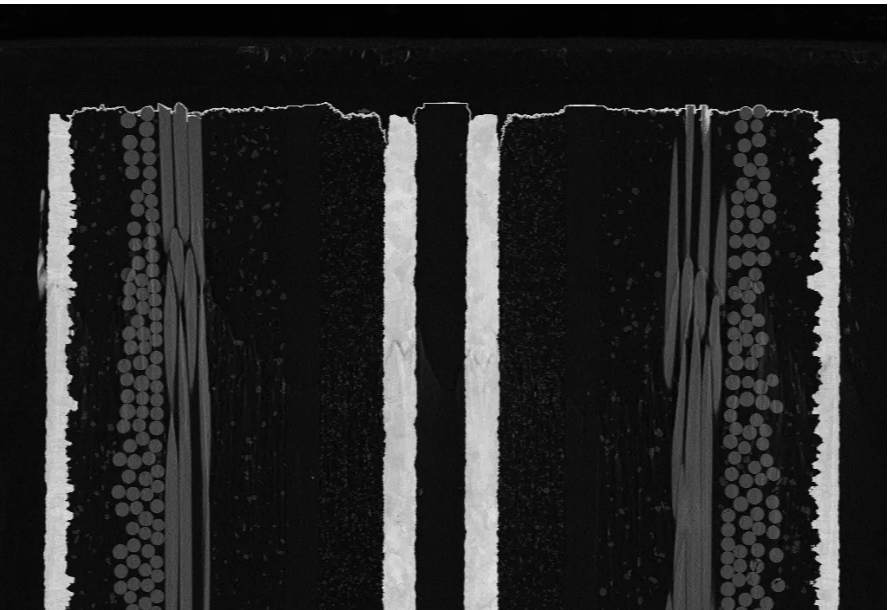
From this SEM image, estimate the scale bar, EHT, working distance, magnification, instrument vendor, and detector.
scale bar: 10000 nm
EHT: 8 kV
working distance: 5.6 mm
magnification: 0.35 K X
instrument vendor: Zeiss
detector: NTS BSD